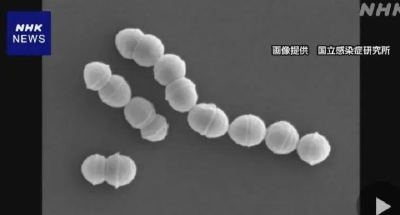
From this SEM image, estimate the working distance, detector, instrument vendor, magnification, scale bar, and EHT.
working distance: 12.1 mm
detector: SE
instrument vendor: Hitachi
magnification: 20 K X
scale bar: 2000 nm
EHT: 5 kV